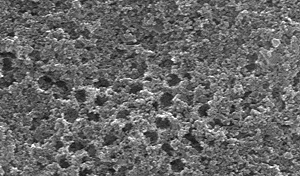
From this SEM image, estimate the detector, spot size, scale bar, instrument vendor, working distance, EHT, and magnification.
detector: SE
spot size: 3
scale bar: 1000 nm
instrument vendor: FEI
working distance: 6.8 mm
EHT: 30 kV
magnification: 65 K X